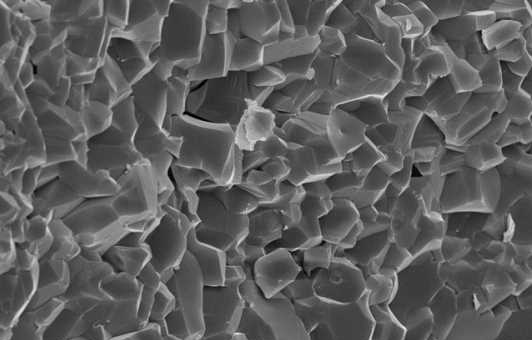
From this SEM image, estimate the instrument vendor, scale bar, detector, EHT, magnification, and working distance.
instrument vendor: Zeiss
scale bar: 2000 nm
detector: InLens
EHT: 15 kV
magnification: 3 K X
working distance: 7.1 mm